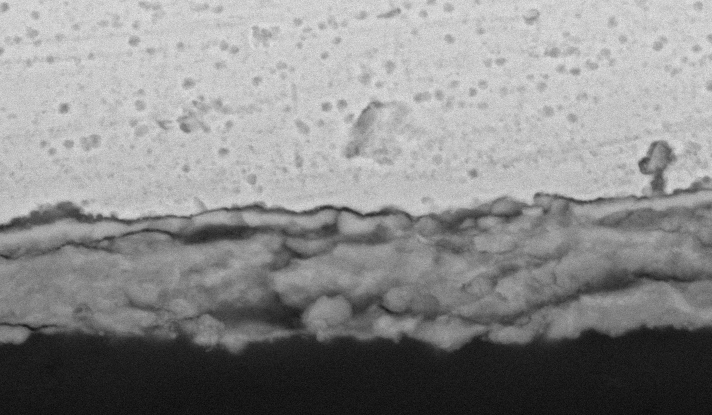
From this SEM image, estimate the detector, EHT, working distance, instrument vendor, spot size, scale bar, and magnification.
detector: BSE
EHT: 20 kV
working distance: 10.2 mm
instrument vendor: FEI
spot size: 4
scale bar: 10000 nm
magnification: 3 K X